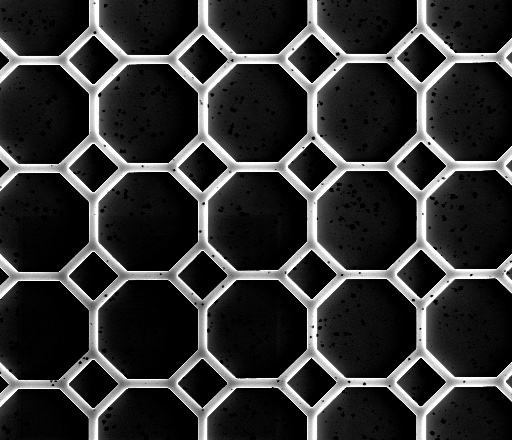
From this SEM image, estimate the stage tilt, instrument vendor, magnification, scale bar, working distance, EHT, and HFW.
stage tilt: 0°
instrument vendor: FEI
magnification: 0.5 K X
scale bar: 50000 nm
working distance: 9.3 mm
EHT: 3.2 kV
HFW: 256 µm